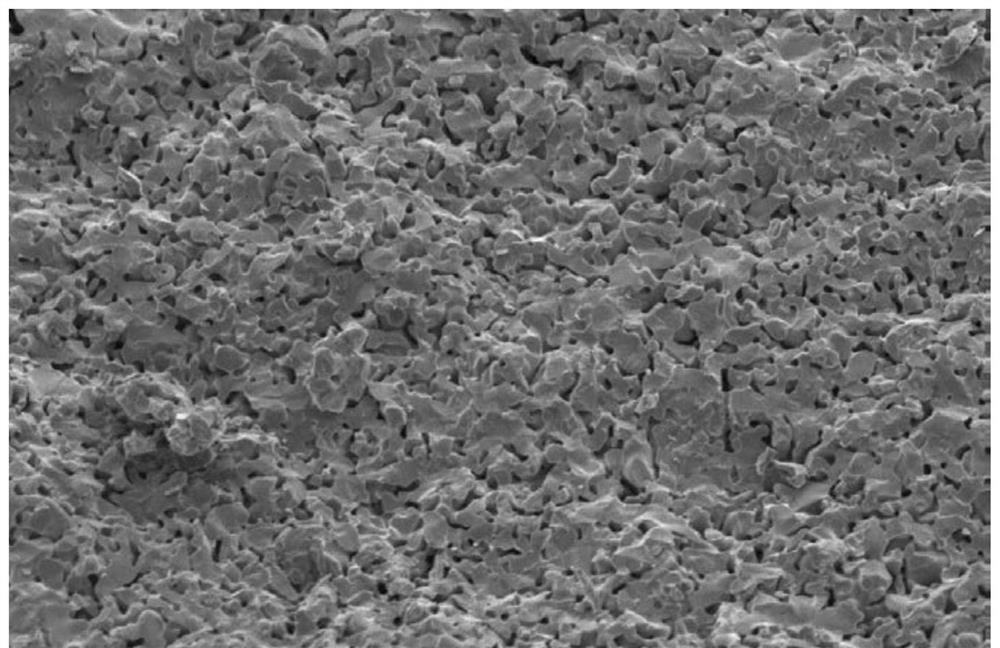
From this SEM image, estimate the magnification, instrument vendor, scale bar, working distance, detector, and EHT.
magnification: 1 K X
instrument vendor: Hitachi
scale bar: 50000 nm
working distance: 12.6 mm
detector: SE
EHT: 5 kV